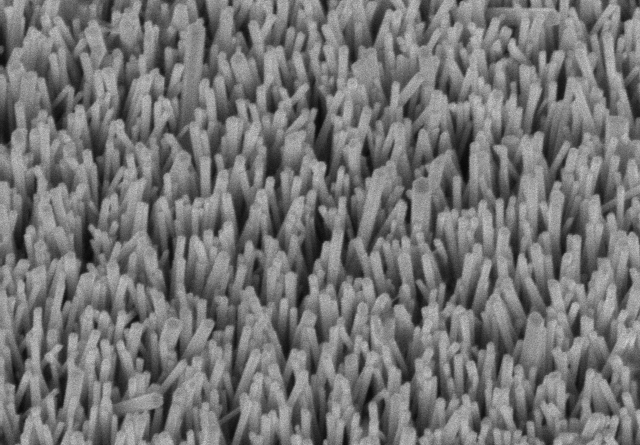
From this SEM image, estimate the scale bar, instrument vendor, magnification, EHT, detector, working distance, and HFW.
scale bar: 1000 nm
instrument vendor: Hitachi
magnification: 30 K X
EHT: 5 kV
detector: SE(U)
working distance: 13.9 mm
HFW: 4.23 µm